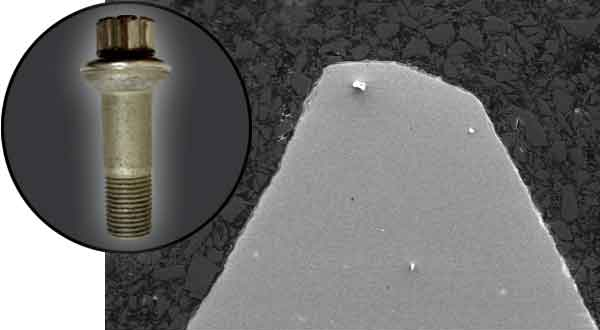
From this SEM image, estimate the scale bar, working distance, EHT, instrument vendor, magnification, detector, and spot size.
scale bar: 100000 nm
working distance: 8.4 mm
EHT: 15 kV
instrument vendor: other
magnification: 0.2 K X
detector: SE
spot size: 8